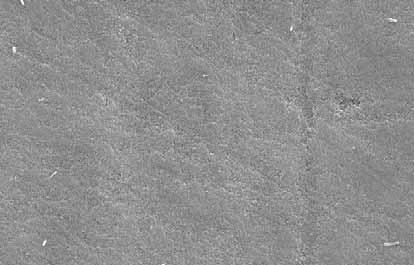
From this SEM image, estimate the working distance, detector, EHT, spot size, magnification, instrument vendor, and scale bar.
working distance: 9.6 mm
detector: SE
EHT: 25 kV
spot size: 3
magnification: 10 K X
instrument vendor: FEI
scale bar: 2000 nm